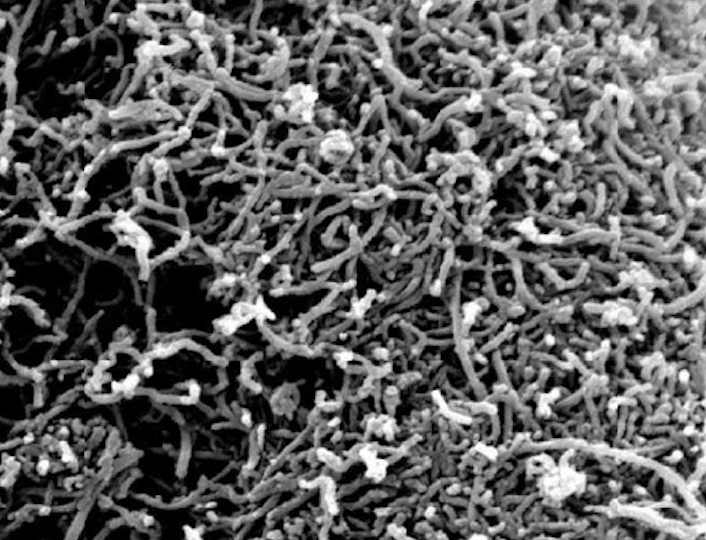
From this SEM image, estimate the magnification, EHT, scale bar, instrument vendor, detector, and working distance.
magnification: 100 K X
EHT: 10 kV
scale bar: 1000 nm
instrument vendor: FEI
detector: SE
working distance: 10.4 mm